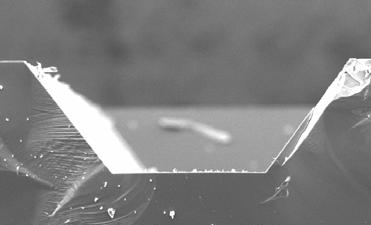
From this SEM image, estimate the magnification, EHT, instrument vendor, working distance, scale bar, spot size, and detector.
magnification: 0.25 K X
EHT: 15 kV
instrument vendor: FEI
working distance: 10 mm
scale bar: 100000 nm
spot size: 3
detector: SE